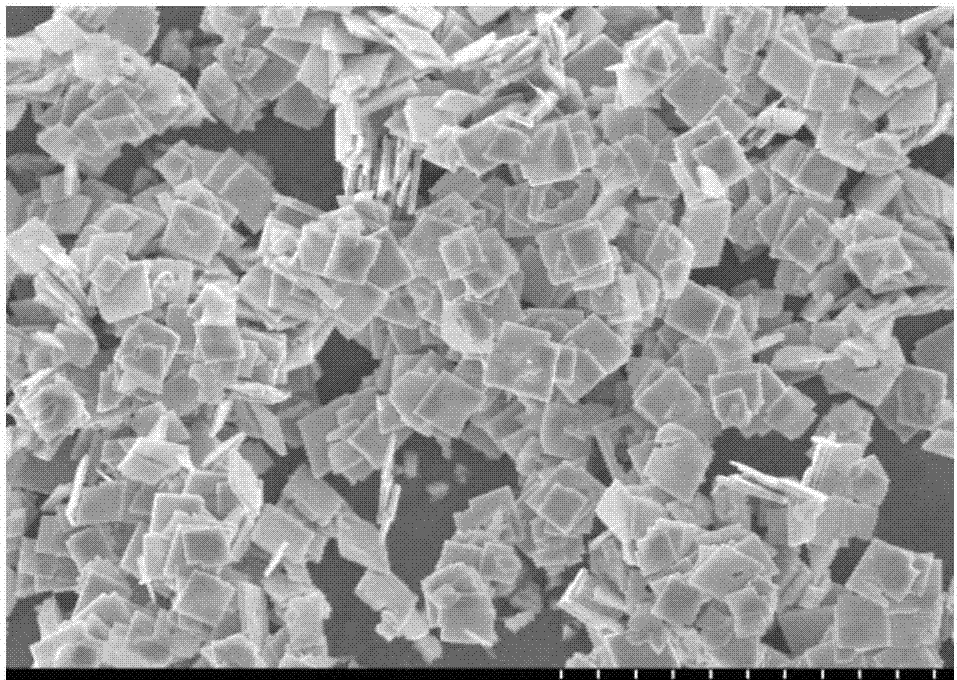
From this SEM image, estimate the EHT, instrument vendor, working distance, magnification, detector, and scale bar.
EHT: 5 kV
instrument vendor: Hitachi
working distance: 7.1 mm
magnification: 10 K X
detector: SE(M)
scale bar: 5000 nm